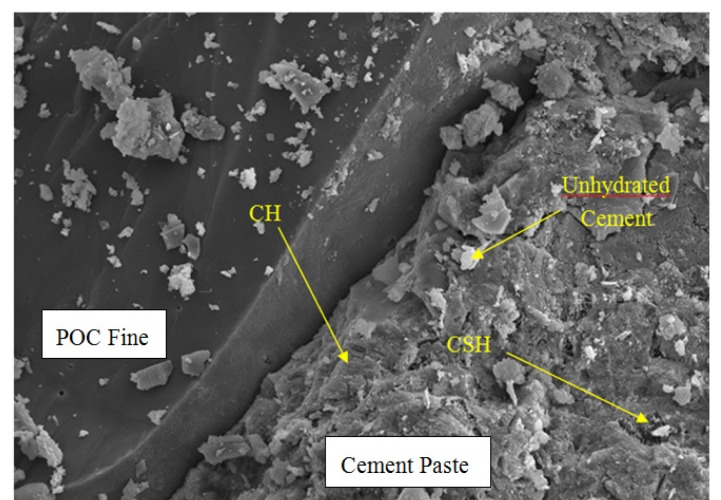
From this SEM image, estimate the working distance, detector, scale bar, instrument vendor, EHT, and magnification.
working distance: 11.4 mm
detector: SE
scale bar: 50000 nm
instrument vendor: Hitachi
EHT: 15 kV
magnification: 1 K X